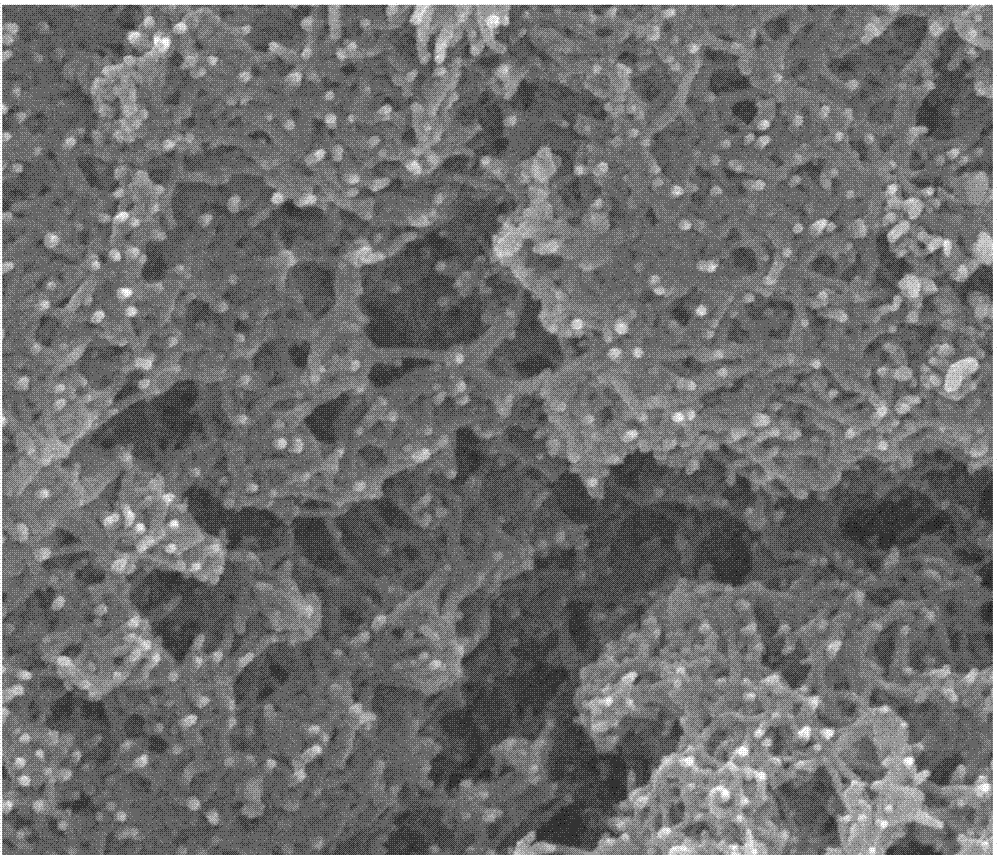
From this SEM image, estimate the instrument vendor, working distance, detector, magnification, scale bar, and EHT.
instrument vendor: FEI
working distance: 9.3 mm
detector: SE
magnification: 100 K X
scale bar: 1000 nm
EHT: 30 kV